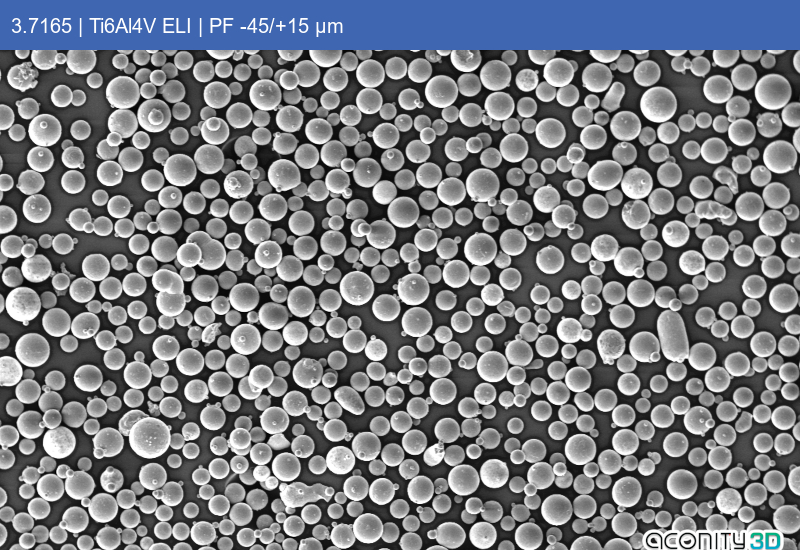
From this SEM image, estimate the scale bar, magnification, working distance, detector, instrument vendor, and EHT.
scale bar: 100000 nm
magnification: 0.1 K X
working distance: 11 mm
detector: SE1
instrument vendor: Zeiss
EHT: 10 kV